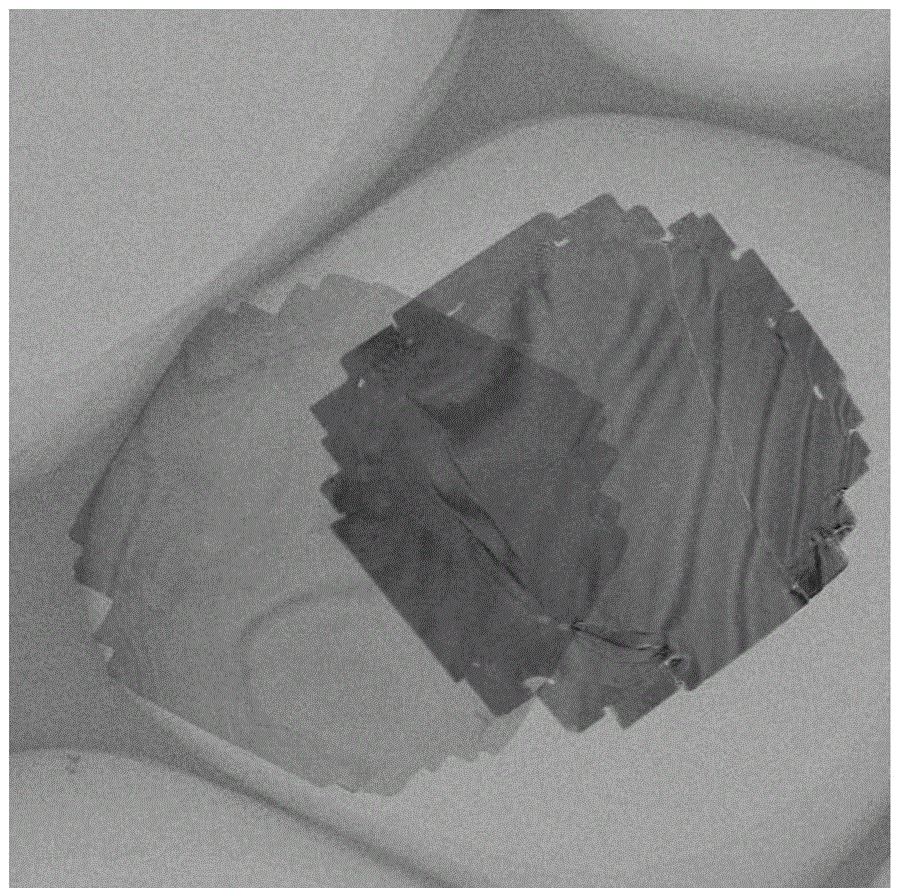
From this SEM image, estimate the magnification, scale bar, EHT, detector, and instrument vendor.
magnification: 80 K X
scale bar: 500 nm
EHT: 200 kV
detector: STEM BF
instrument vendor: JEOL